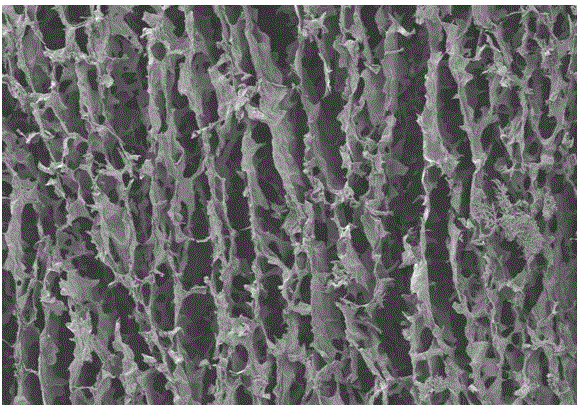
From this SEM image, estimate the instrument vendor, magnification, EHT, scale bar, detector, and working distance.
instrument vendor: Hitachi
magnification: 0.12 K X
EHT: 3 kV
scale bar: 400000 nm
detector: SE(M)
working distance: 8.6 mm